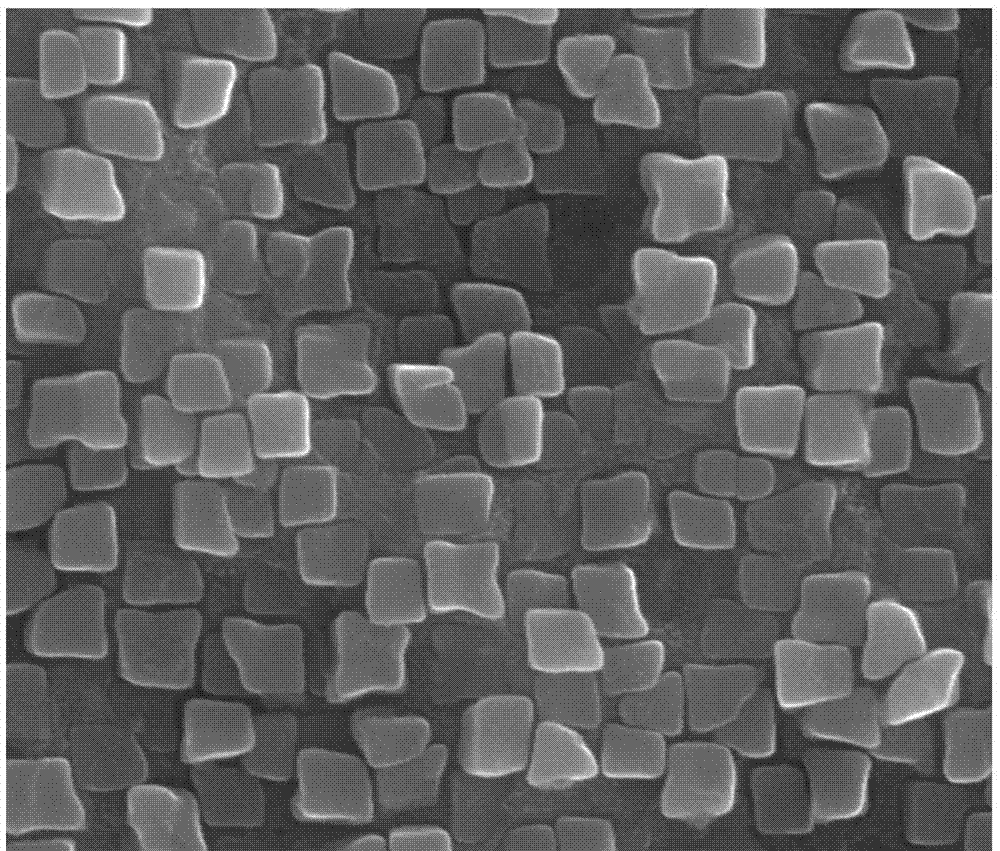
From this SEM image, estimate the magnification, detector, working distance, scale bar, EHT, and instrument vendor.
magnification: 80 K X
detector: ETD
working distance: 12.1 mm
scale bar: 1000 nm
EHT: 30 kV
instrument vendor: FEI